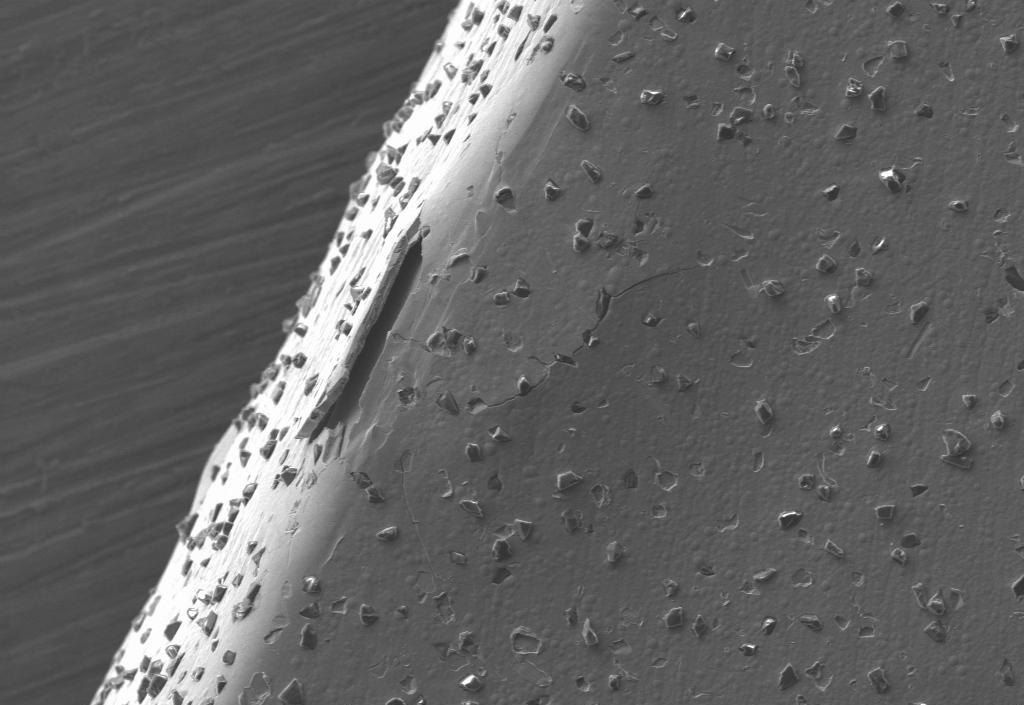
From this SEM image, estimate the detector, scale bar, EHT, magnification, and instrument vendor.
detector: SE1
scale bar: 20000 nm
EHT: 15 kV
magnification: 0.5 K X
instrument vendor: Zeiss